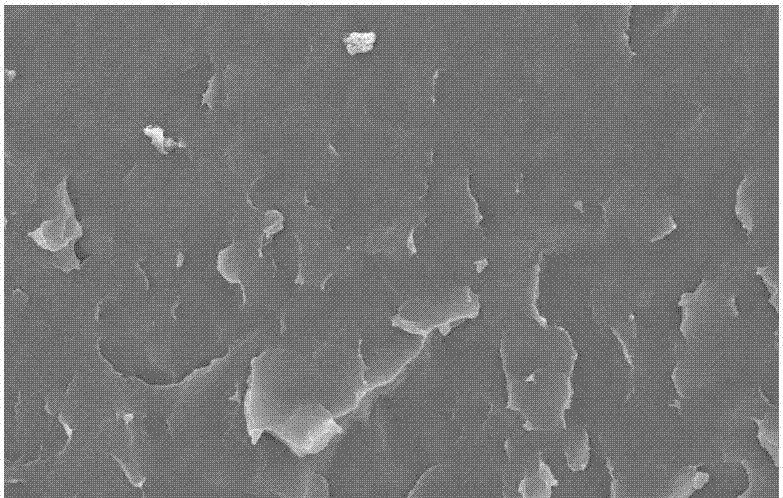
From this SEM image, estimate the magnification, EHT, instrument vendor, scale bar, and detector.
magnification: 1 K X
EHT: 20 kV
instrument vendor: JEOL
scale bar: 10000 nm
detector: SEI